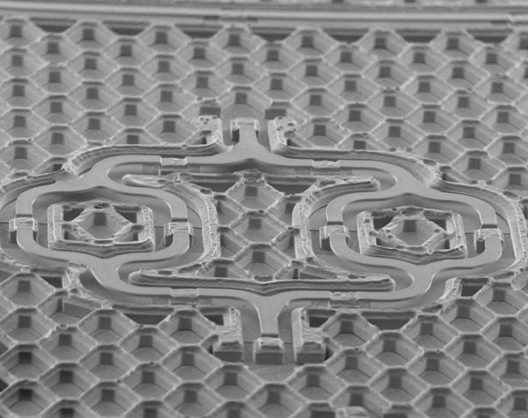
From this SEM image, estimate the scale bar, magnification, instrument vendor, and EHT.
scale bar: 500000 nm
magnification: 0.06 K X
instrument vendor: JEOL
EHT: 10 kV